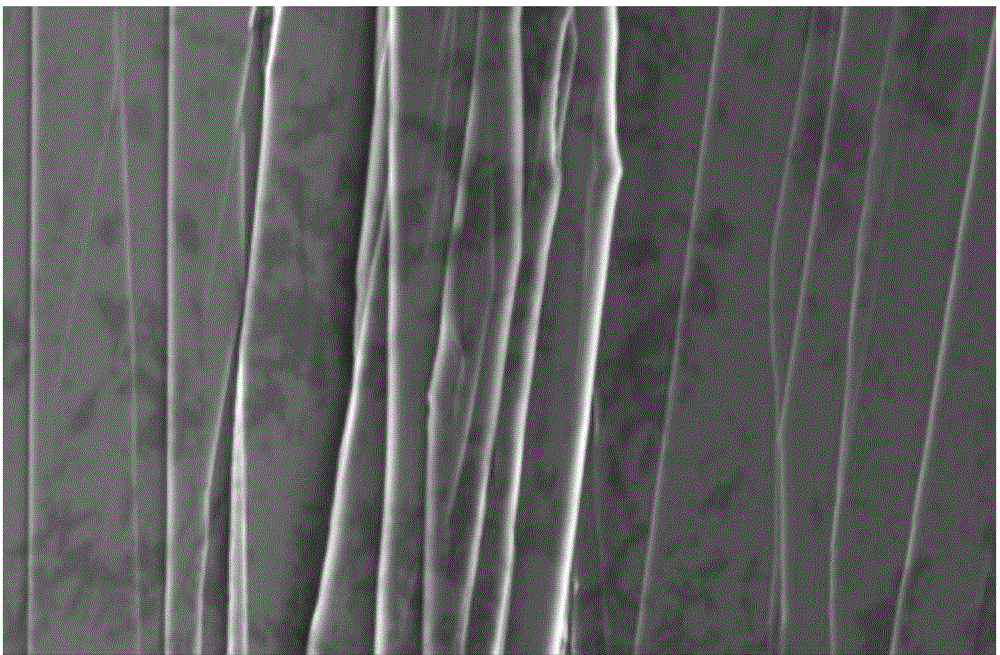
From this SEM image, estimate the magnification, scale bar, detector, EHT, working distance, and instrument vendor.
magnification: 2 K X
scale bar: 20000 nm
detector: SE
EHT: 15 kV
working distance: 15 mm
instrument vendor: Hitachi